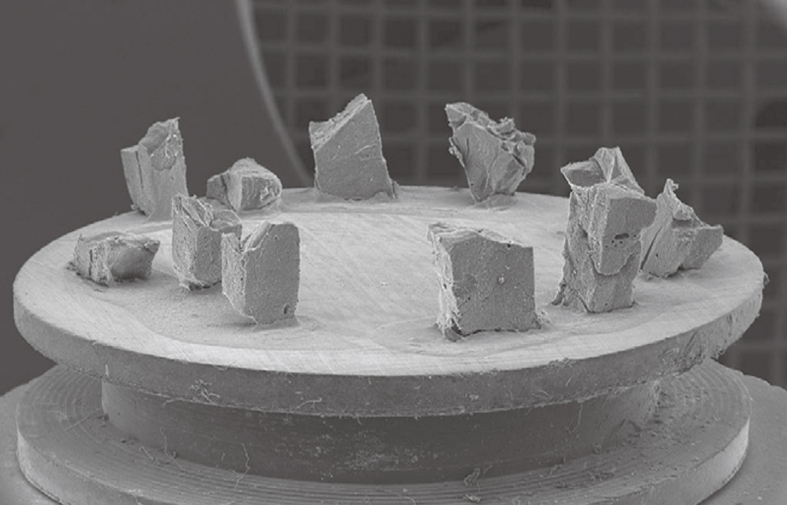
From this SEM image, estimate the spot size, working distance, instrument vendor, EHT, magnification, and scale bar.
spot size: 3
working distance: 34.3 mm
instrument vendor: FEI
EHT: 30 kV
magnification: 0.018 K X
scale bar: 2000000 nm